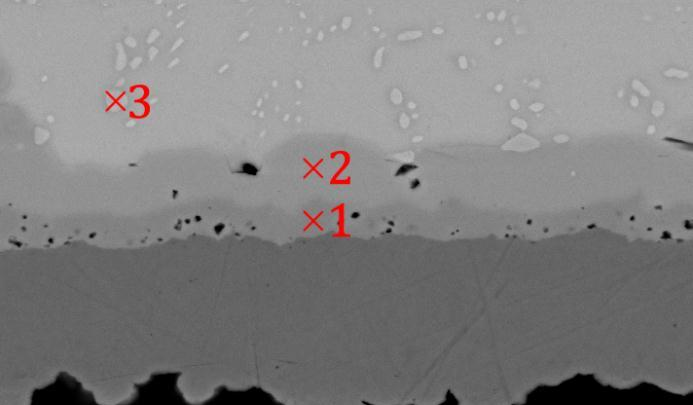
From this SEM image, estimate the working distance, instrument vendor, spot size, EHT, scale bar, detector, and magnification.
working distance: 11.7 mm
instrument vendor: FEI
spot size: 3.5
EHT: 20 kV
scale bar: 10000 nm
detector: BSE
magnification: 2 K X